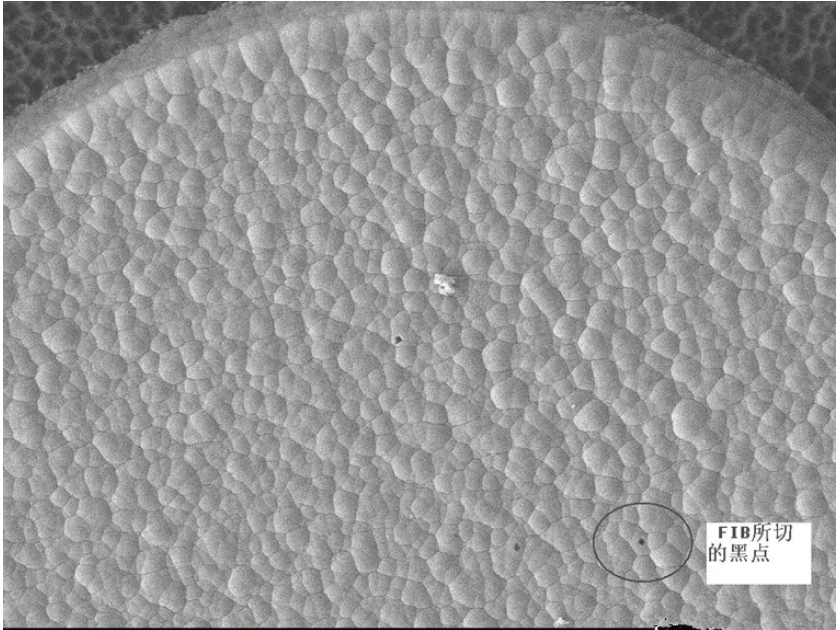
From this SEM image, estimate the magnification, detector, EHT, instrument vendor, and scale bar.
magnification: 0.8 K X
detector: LEI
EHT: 10 kV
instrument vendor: JEOL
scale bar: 10000 nm